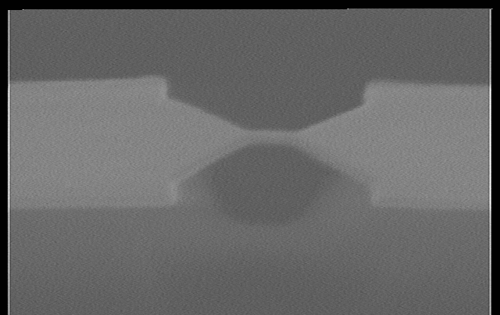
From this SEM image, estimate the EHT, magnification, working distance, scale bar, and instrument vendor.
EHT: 25 kV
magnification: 25 K X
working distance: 39 mm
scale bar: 1000 nm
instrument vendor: JEOL